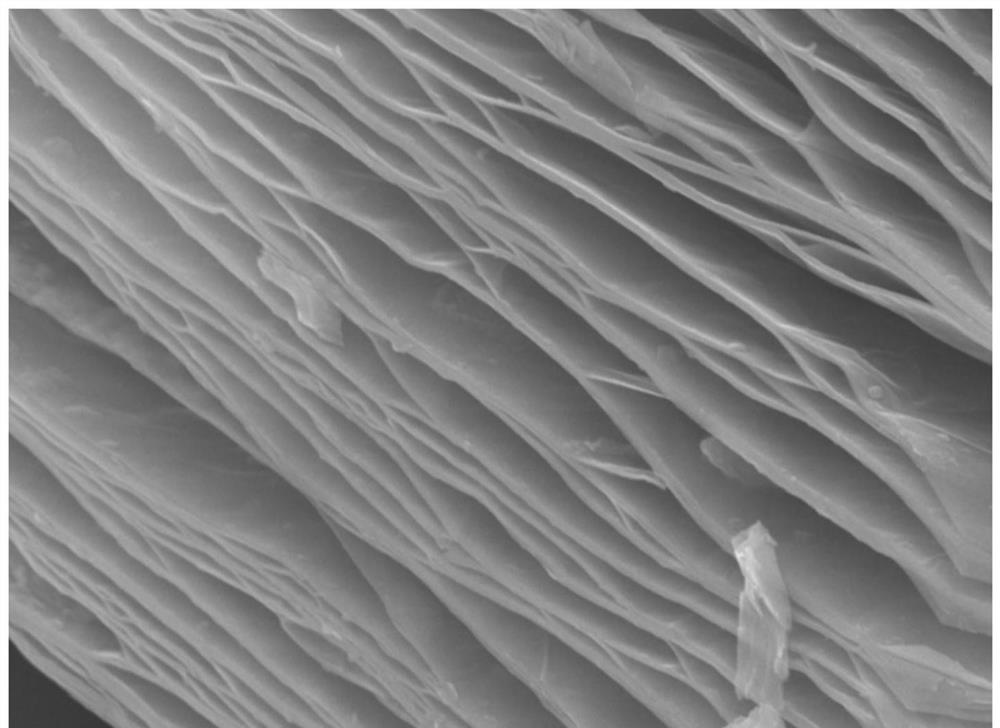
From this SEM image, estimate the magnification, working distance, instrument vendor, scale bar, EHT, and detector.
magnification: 20 K X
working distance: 10.6 mm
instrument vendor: JEOL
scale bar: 1000 nm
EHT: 15 kV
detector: SEI